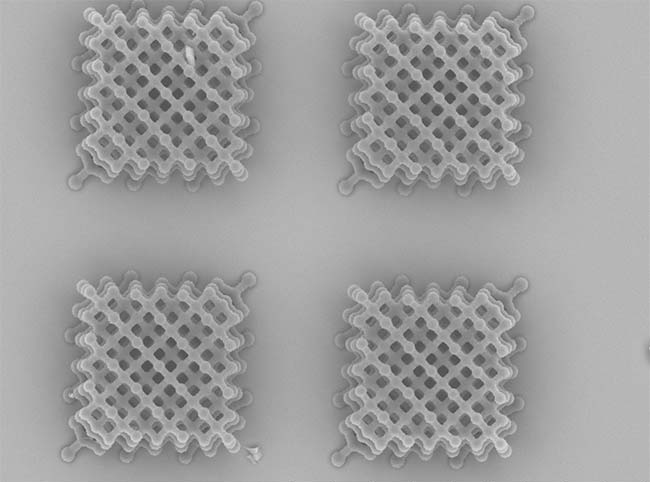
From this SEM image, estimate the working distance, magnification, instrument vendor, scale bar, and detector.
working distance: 9.5 mm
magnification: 1 K X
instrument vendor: Hitachi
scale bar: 100000 nm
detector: N